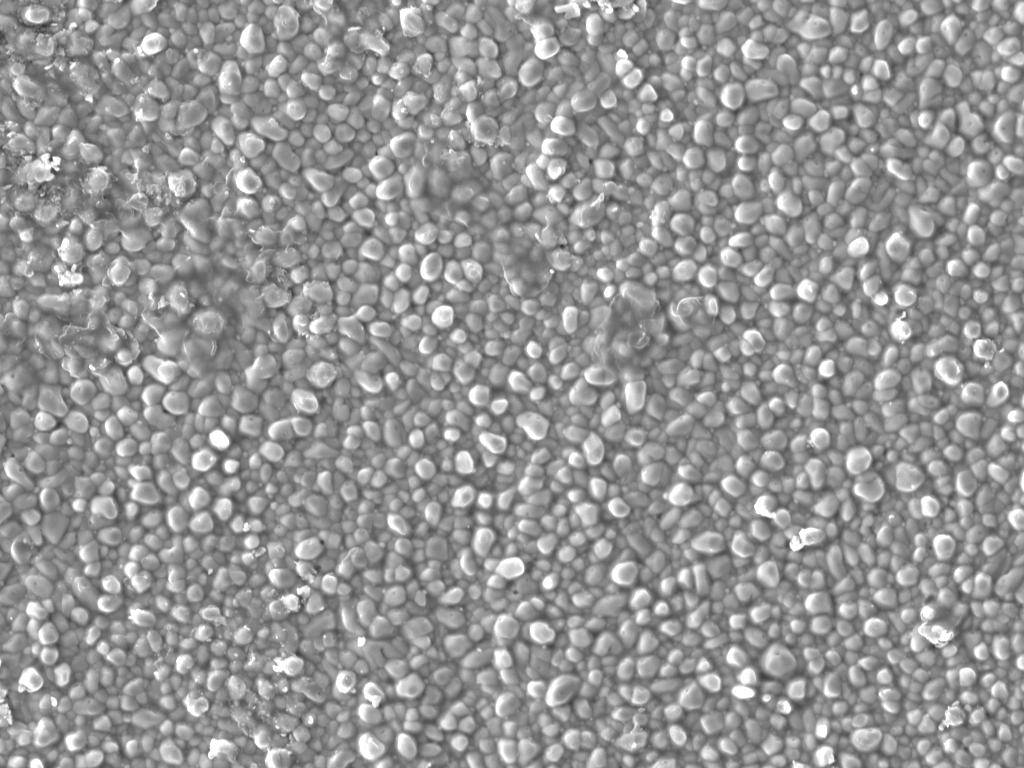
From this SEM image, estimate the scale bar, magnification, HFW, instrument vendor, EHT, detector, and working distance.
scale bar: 20000 nm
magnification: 2.5 K X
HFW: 111 µm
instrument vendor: Tescan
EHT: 20 kV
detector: SE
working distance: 19.88 mm